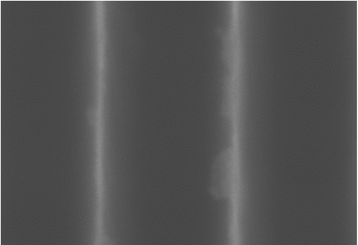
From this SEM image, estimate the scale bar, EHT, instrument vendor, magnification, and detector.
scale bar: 100 nm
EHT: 10 kV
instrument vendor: Hitachi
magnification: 350 K X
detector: SE(U)